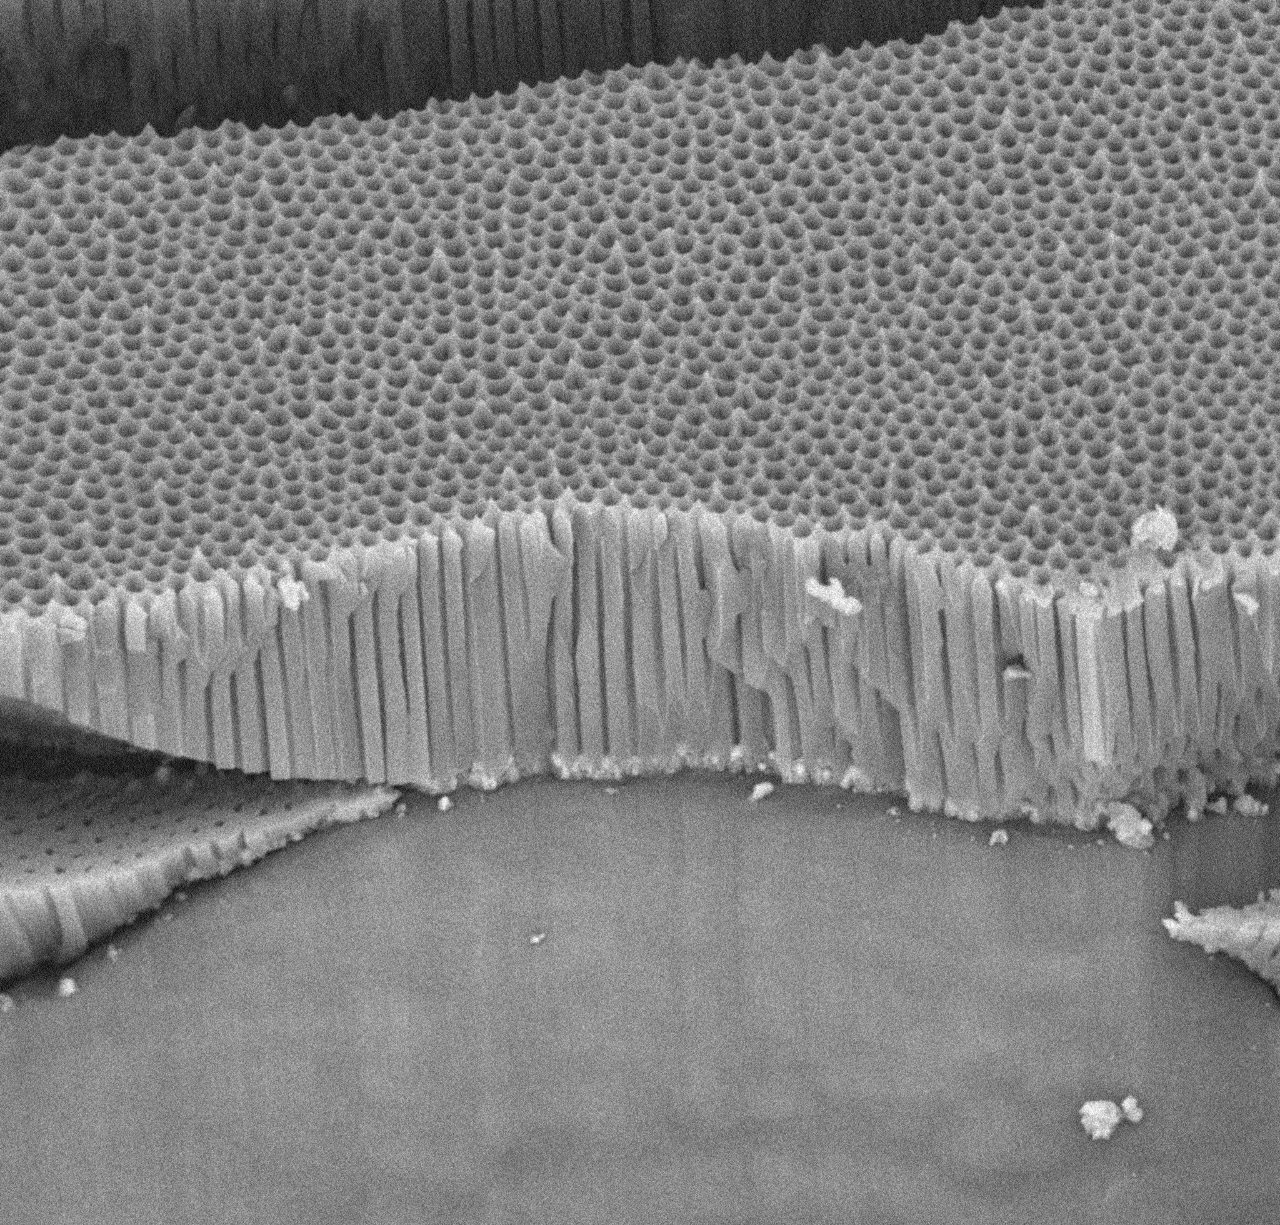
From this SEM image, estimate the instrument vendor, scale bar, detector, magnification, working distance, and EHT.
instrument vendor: other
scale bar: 5000 nm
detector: BSE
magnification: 10 K X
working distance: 6.5 mm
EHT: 15 kV